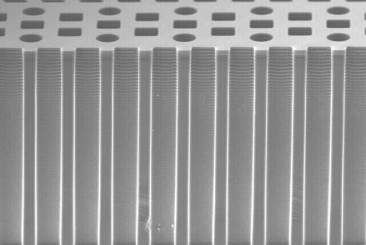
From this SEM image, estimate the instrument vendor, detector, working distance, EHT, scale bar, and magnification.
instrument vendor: Hitachi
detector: SE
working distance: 4.2 mm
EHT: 20 kV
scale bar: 200000 nm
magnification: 0.25 K X